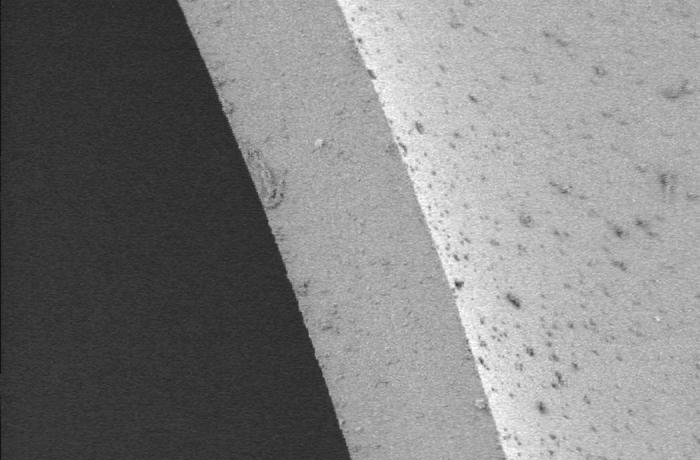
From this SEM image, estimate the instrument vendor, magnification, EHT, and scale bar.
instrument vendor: Hitachi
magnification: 0.5 K X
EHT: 5 kV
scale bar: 50000 nm